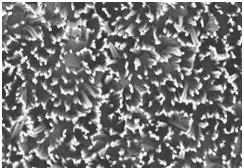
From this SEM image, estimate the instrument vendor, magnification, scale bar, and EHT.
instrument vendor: Hitachi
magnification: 20 K X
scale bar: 2000 nm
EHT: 1.8 kV